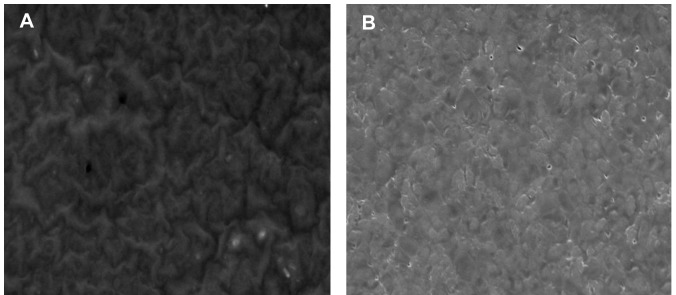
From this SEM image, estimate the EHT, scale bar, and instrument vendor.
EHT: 20 kV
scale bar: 10000 nm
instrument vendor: JEOL